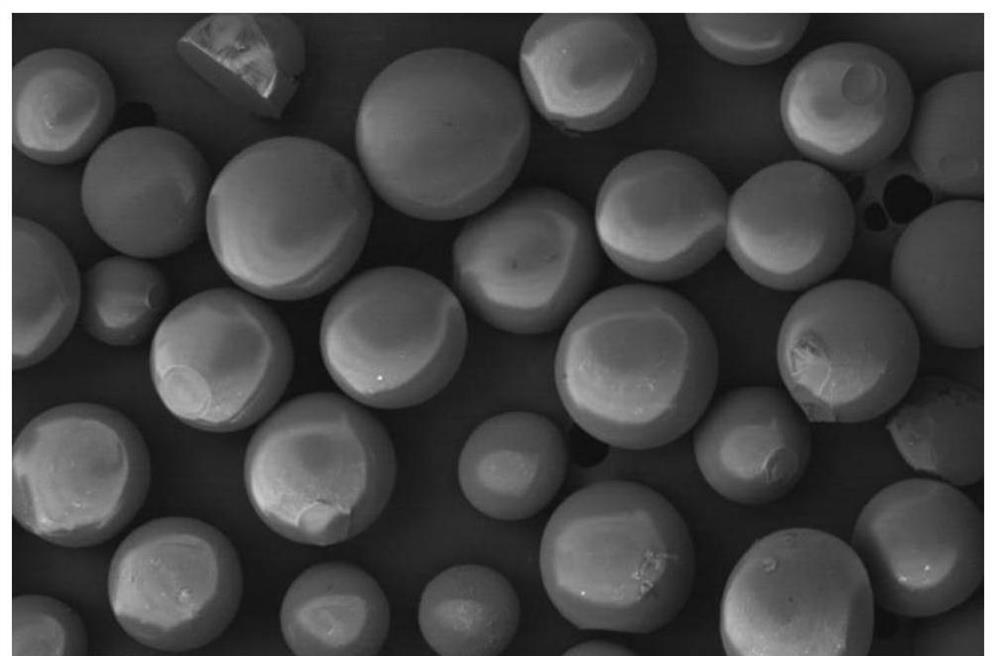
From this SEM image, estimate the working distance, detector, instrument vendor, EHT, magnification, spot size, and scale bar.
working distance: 4.3 mm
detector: CBS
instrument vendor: FEI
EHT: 10 kV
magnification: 0.14 K X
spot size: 3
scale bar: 300000 nm